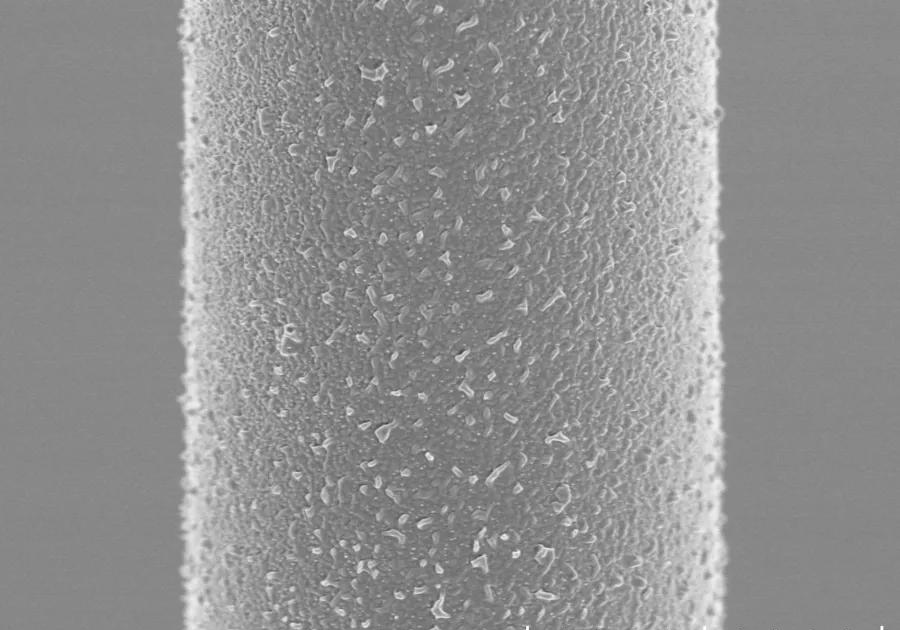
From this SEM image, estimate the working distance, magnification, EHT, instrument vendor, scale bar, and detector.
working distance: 5.1 mm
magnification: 10 K X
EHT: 2 kV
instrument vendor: Hitachi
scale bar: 5000 nm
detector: SE(UL)